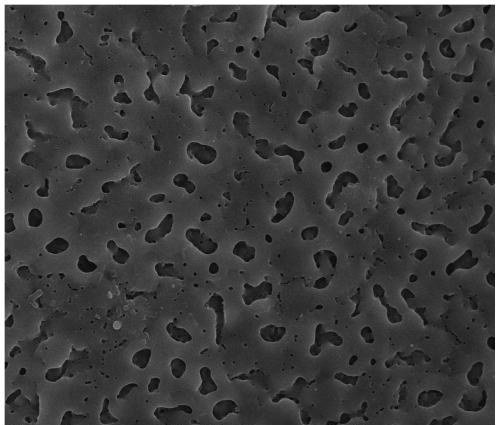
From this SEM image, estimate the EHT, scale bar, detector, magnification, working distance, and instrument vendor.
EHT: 15 kV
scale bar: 10000 nm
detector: TLD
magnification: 10 K X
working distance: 5.1 mm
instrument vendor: FEI